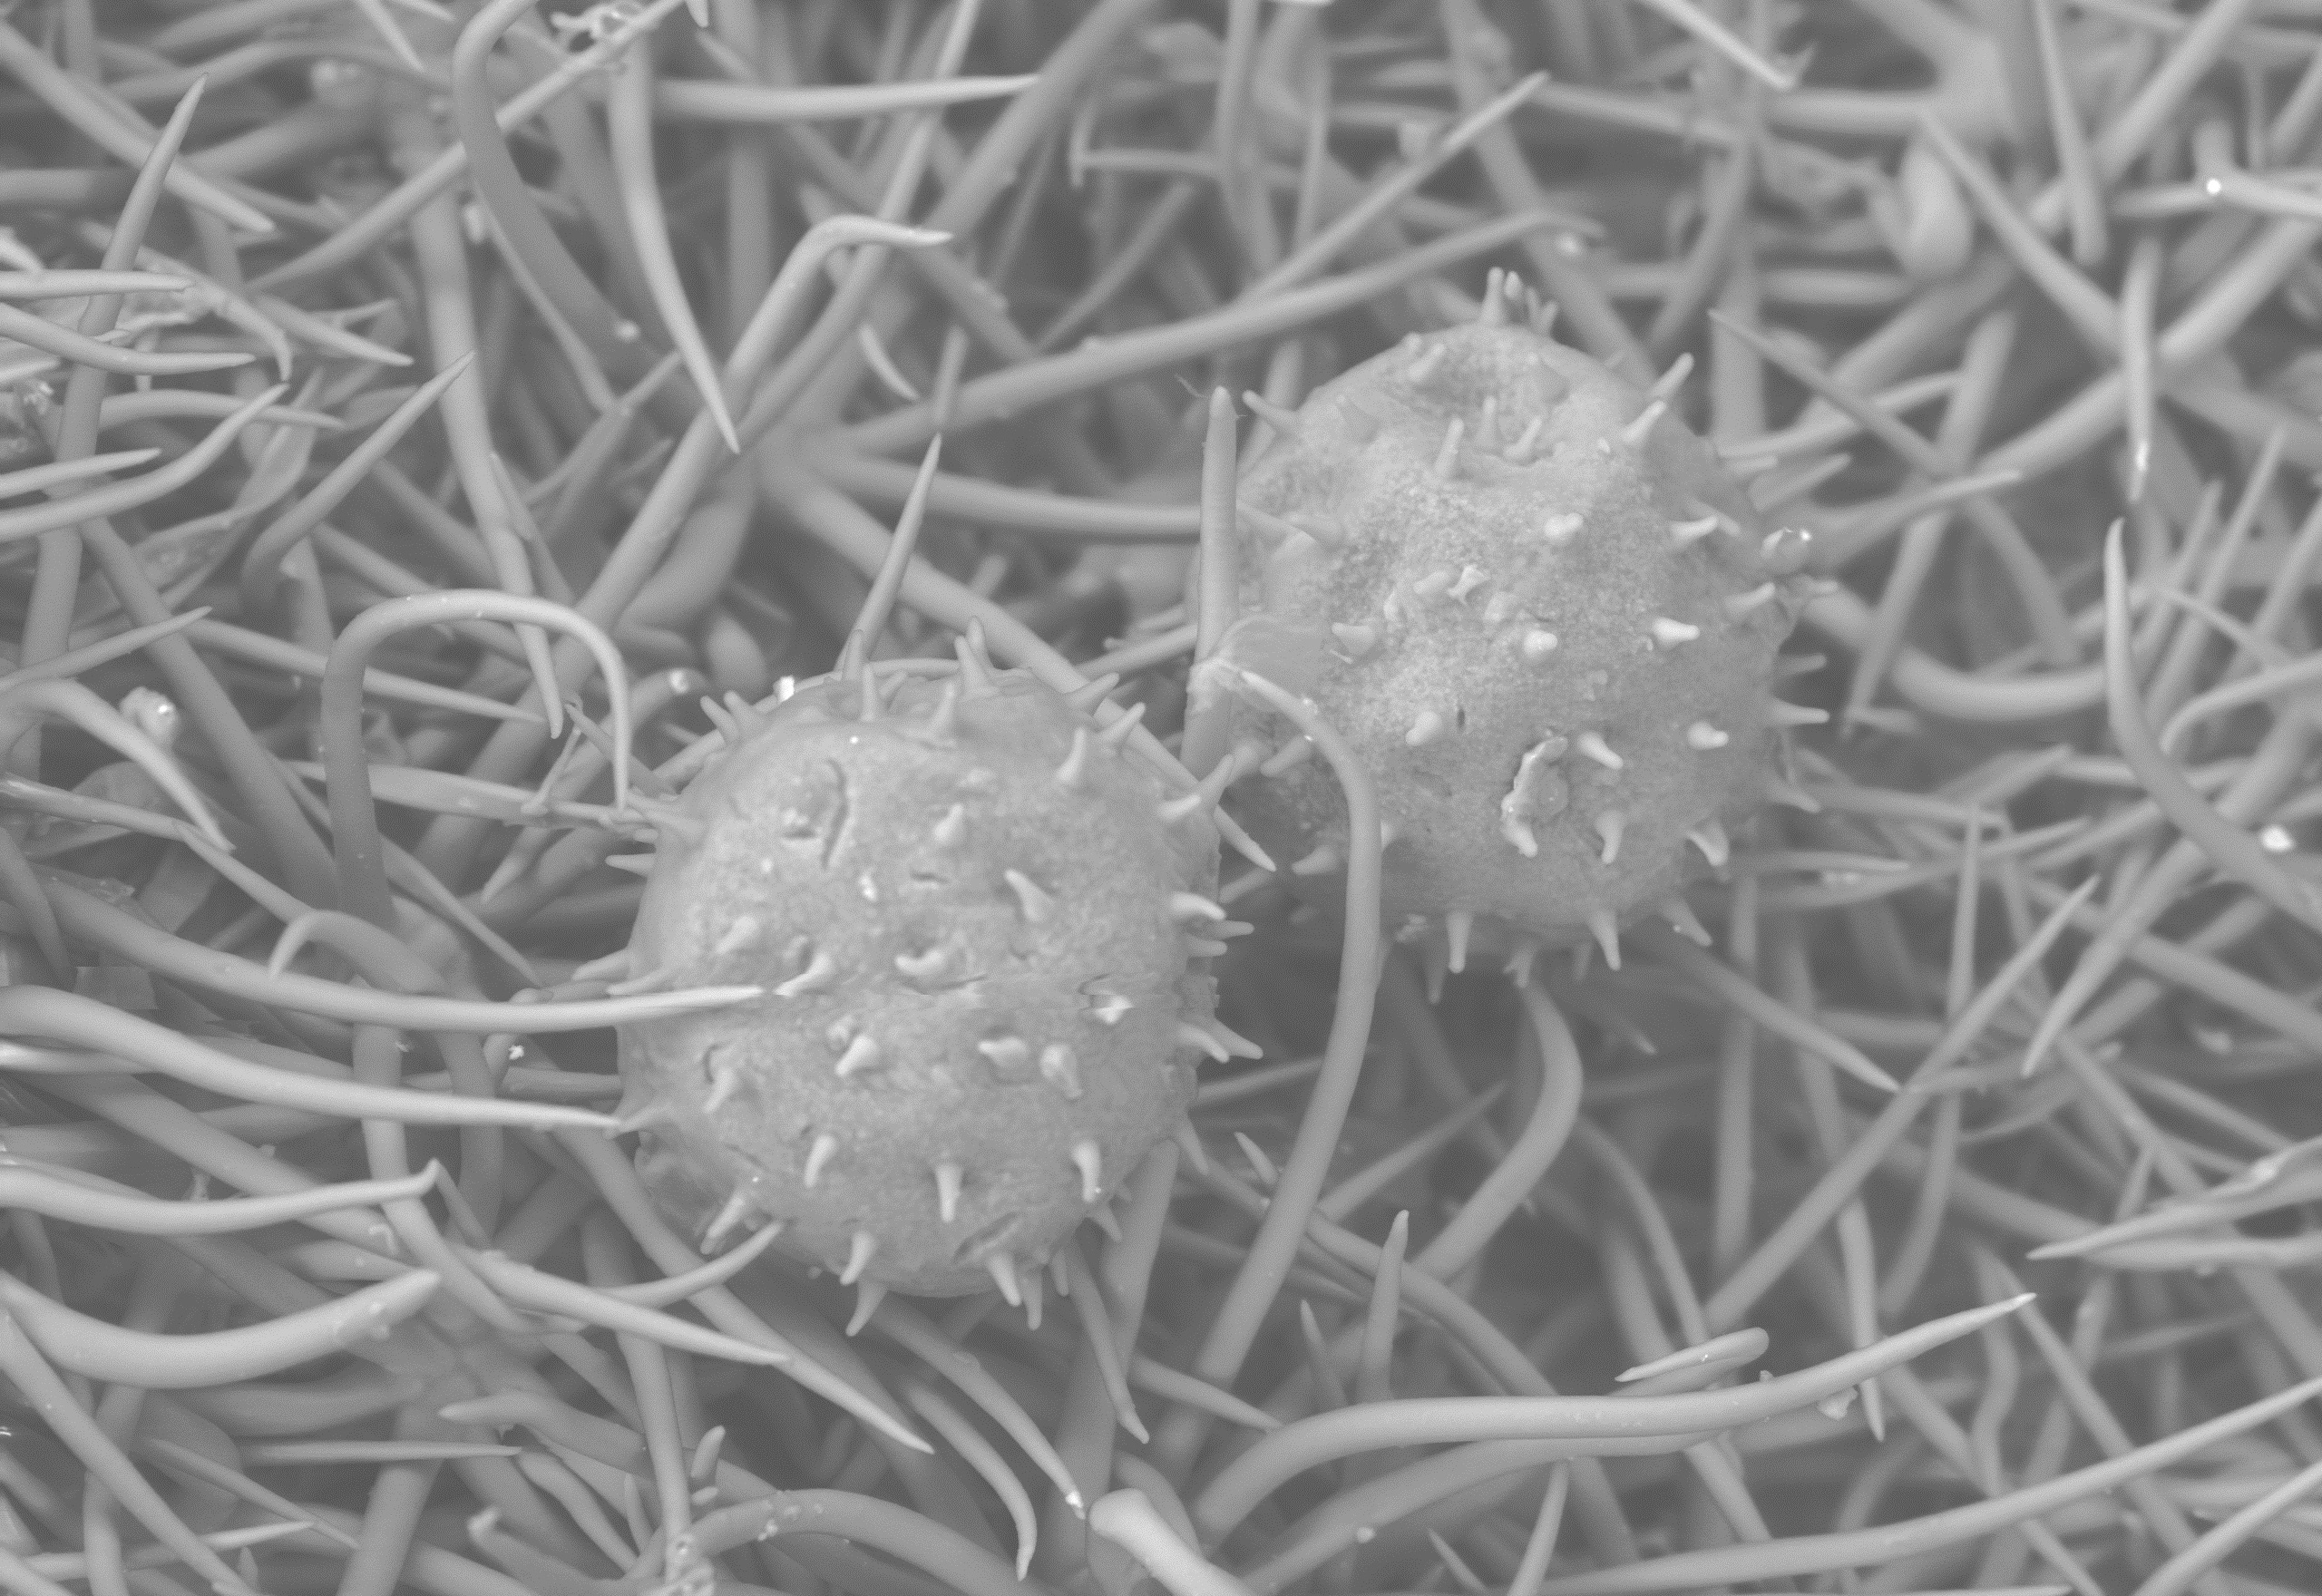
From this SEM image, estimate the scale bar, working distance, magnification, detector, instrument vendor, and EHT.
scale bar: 100000 nm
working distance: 11.1 mm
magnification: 0.3 K X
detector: BSE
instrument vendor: Hitachi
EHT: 15 kV